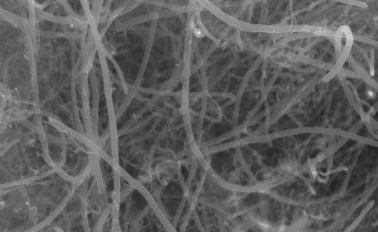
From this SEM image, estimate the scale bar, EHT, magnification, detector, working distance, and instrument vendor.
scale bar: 1000 nm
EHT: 15 kV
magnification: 30 K X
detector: SE(M,-150)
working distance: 12.2 mm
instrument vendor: Hitachi